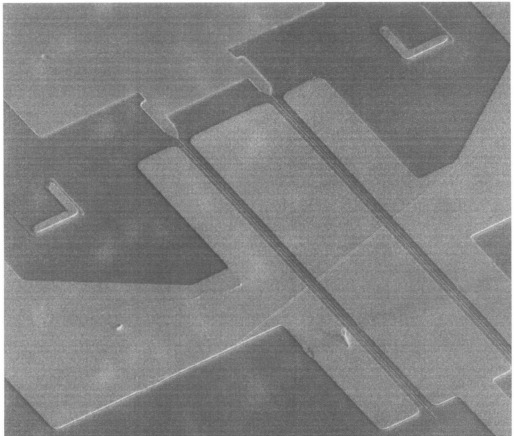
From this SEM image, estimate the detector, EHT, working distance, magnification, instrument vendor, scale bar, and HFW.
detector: SE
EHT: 10 kV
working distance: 4.1 mm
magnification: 2.5 K X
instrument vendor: FEI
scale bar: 30000 nm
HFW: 102 µm